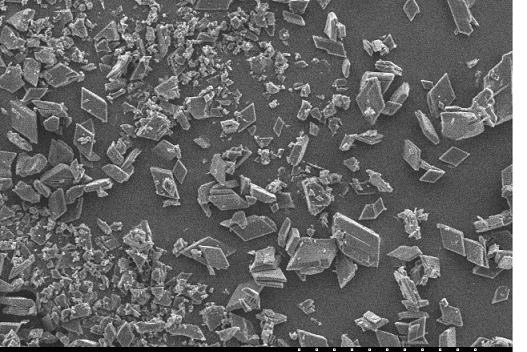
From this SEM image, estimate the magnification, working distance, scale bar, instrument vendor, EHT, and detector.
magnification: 0.15 K X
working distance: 22.1 mm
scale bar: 300000 nm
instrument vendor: JEOL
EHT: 15 kV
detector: SE